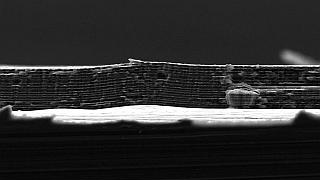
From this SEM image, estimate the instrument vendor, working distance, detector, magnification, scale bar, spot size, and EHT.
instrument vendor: FEI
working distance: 10 mm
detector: SE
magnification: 3.198 K X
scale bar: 10000 nm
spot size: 2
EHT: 5 kV